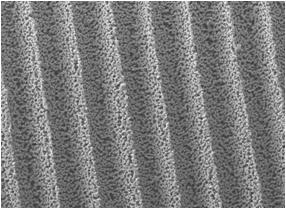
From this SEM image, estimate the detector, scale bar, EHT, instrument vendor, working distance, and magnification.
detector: LEI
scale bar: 10000 nm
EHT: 10 kV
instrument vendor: JEOL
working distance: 8 mm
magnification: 0.8 K X